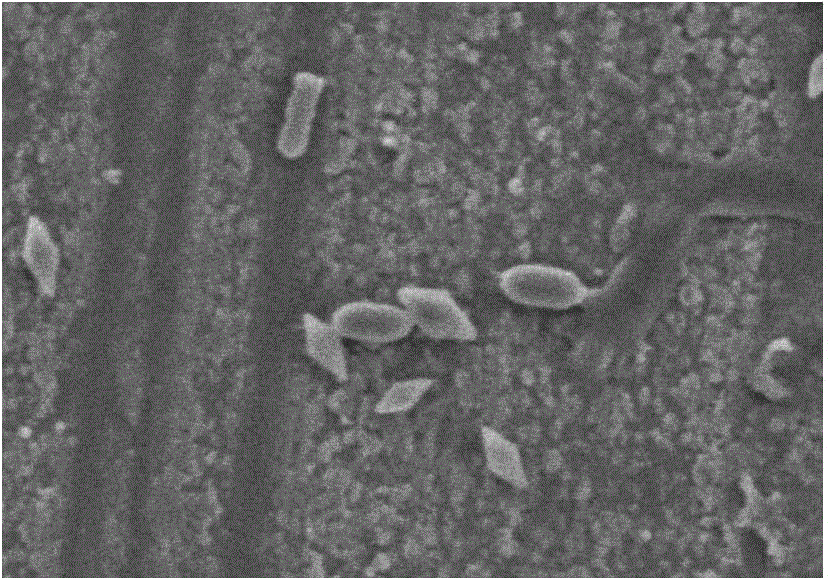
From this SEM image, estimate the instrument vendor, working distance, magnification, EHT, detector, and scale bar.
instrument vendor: Hitachi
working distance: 10.8 mm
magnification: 7.5 K X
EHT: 5 kV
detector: SE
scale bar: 5000 nm